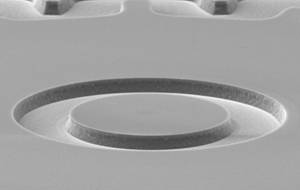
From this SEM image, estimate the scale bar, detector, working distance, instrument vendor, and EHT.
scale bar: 20000 nm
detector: SE1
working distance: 28 mm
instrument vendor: Zeiss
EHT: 15 kV